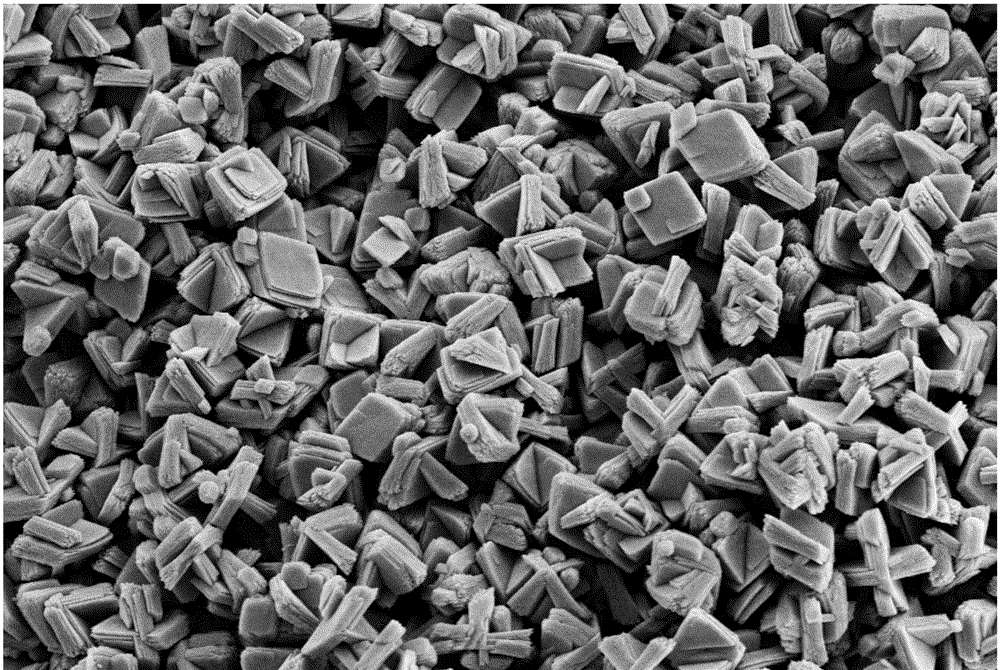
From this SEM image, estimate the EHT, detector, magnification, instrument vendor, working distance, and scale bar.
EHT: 5 kV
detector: SE2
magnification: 5 K X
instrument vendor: Zeiss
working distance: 8.9 mm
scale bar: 2000 nm